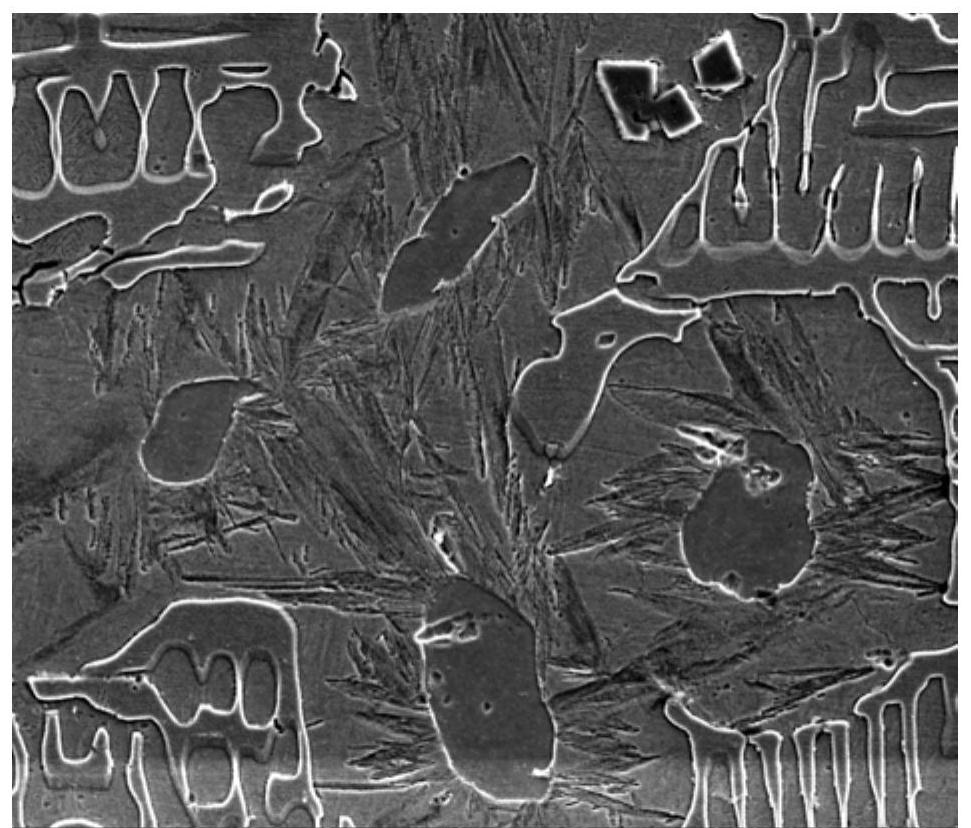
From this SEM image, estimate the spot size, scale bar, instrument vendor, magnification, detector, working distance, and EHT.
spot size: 3.5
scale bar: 10000 nm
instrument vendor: FEI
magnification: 5 K X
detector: ETD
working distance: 8.2 mm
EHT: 10 kV